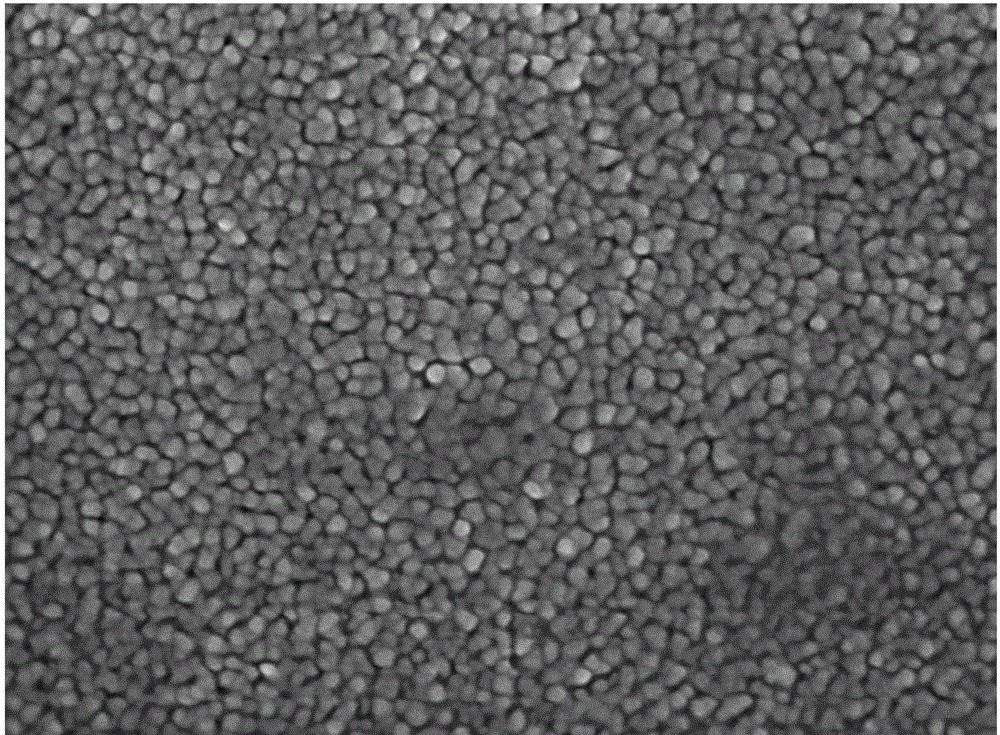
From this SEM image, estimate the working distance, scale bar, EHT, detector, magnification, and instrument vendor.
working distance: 7.1 mm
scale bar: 100 nm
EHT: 5 kV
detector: SEI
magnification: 65 K X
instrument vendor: JEOL